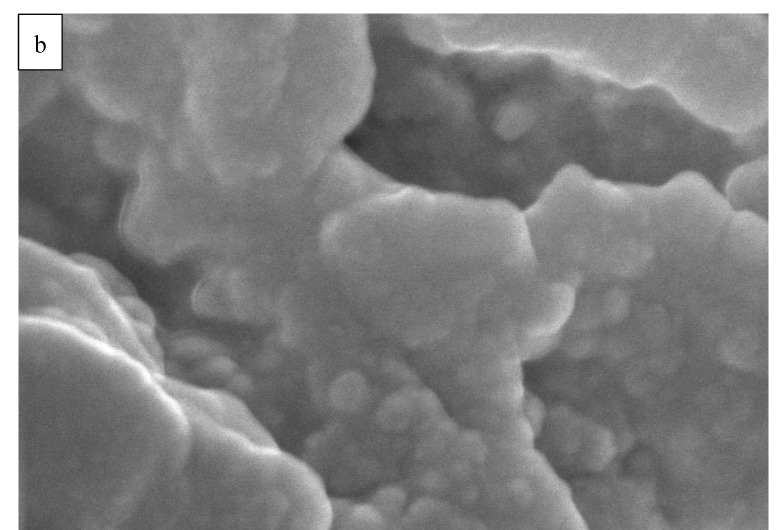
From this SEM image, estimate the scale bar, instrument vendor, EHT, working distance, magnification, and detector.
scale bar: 300 nm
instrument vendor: Hitachi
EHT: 10 kV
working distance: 9 mm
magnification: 150 K X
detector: SE(U,LA0)